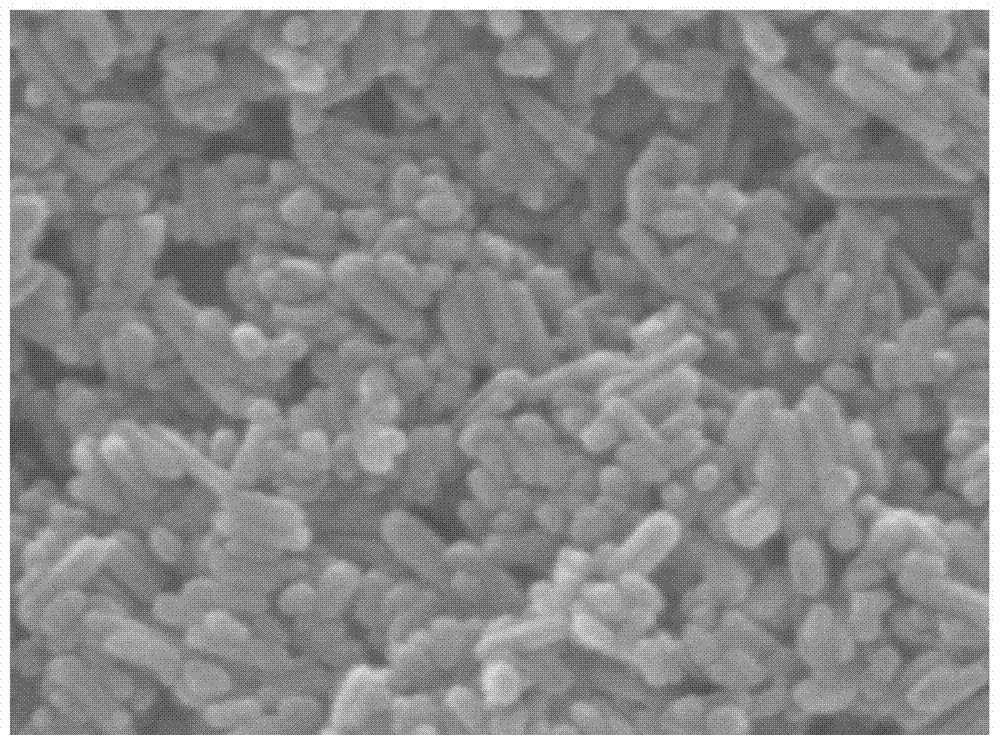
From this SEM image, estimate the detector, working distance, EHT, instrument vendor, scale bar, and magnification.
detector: SEI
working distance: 10.2 mm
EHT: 15 kV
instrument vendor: JEOL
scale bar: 100 nm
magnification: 100 K X